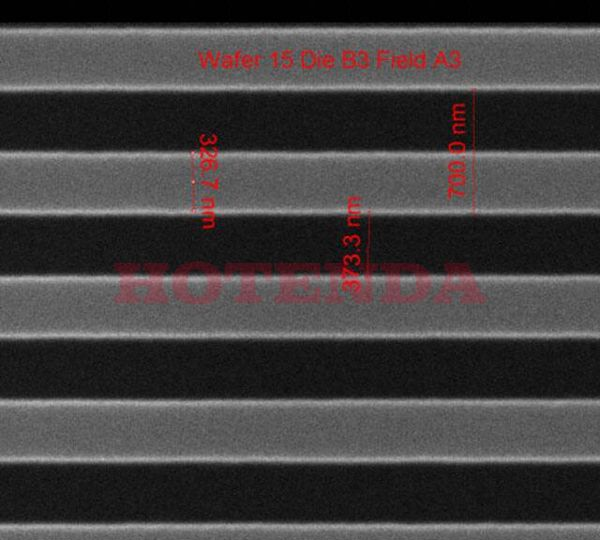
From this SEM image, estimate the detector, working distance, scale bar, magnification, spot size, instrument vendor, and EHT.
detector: ETD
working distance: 10.2 mm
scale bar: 1000 nm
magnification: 75.019 K X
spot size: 1.5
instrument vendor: FEI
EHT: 10 kV